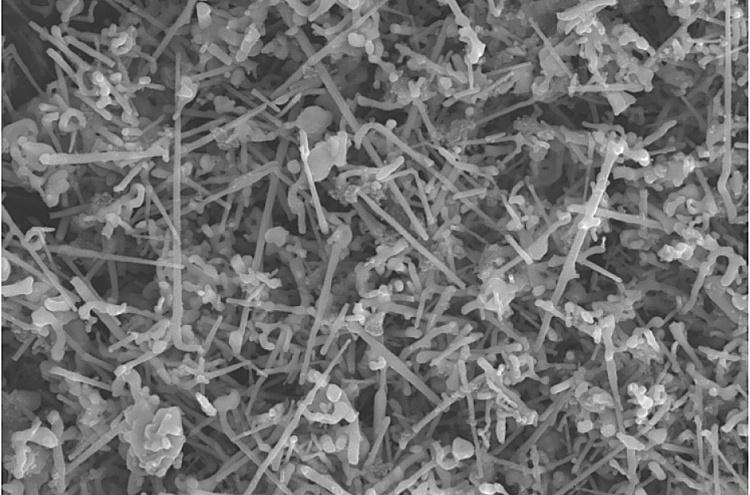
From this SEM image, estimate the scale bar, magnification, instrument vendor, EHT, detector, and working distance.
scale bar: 2000 nm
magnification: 2 K X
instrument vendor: Zeiss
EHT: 21 kV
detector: SE2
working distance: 8.1 mm